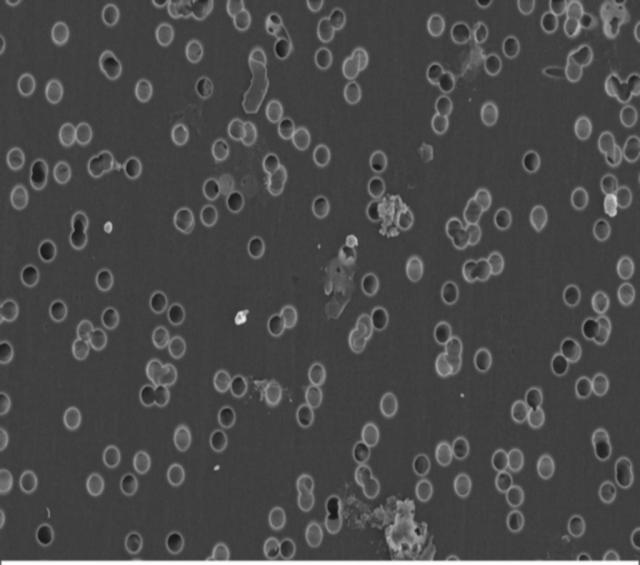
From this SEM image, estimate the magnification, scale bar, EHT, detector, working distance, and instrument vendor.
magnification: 10 K X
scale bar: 1000 nm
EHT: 3 kV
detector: InLens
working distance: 2.9 mm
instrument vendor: Zeiss